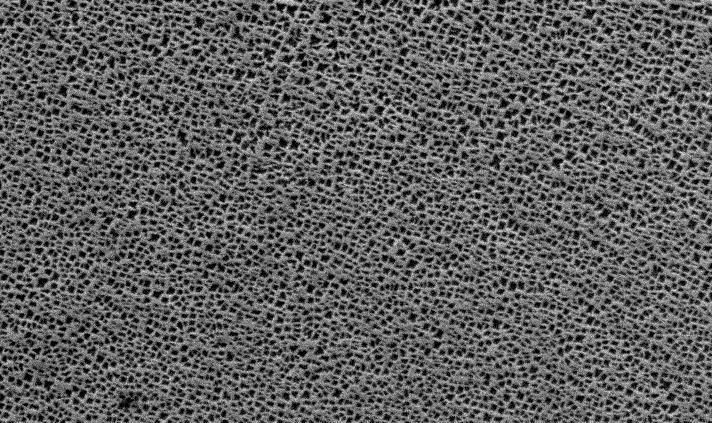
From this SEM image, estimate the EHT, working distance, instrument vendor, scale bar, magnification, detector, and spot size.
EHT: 20 kV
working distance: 10.6 mm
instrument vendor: FEI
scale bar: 10000 nm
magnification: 2.5 K X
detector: SE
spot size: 4